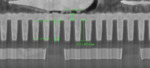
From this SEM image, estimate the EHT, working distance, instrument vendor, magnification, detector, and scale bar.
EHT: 2 kV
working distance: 5 mm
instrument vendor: Zeiss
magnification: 45 K X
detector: SE2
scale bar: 1000 nm